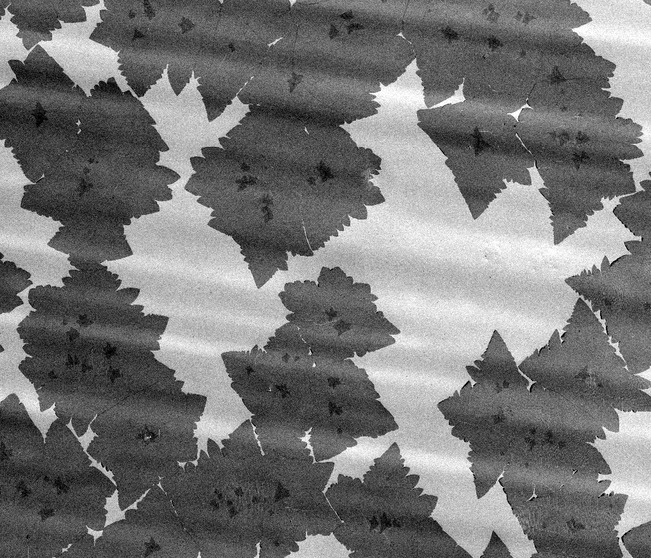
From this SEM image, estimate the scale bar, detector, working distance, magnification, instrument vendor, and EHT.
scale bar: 50000 nm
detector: ETD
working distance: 4.1 mm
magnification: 0.5 K X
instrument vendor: FEI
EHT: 5 kV